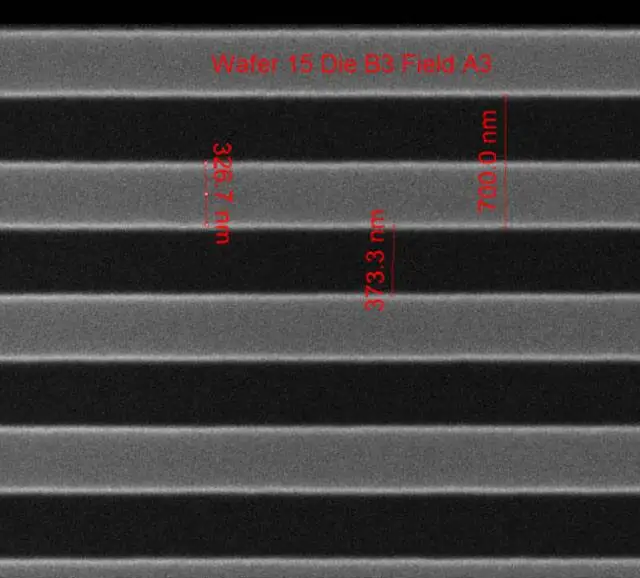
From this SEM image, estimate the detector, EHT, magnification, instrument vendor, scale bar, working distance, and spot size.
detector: ETD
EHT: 10 kV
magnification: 75.019 K X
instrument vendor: FEI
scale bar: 1000 nm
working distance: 10.2 mm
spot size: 1.5